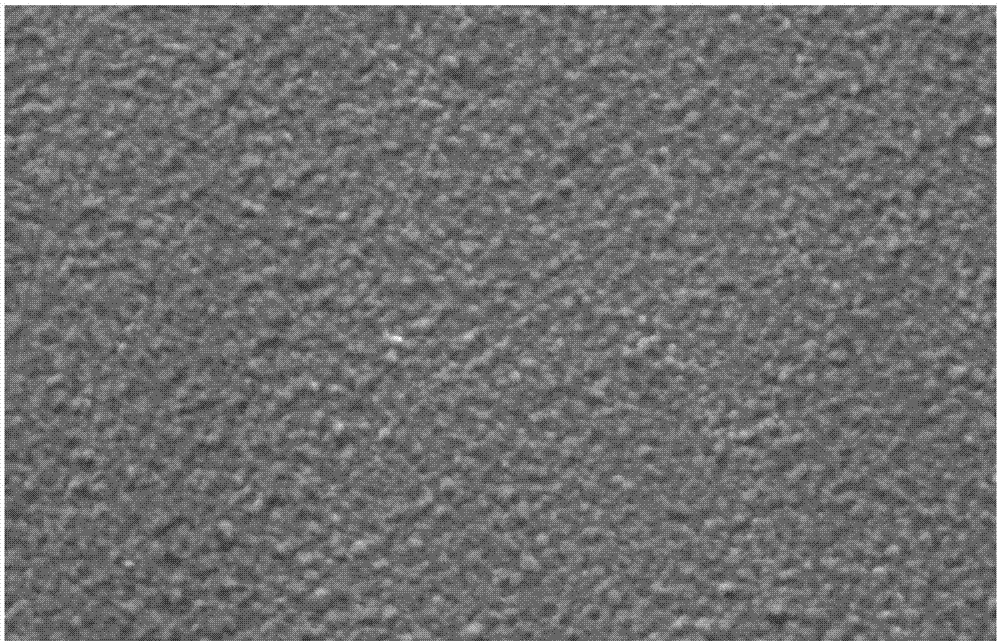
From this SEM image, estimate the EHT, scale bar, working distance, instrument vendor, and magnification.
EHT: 15 kV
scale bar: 2000 nm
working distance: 10.5 mm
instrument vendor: Zeiss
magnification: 5 K X